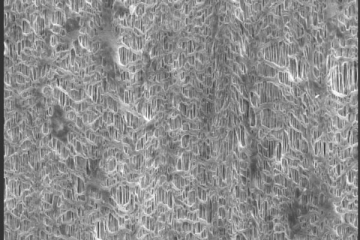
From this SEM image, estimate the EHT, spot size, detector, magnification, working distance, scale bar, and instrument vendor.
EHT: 10 kV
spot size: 3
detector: SE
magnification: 5 K X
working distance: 11.9 mm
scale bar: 5000 nm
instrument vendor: FEI